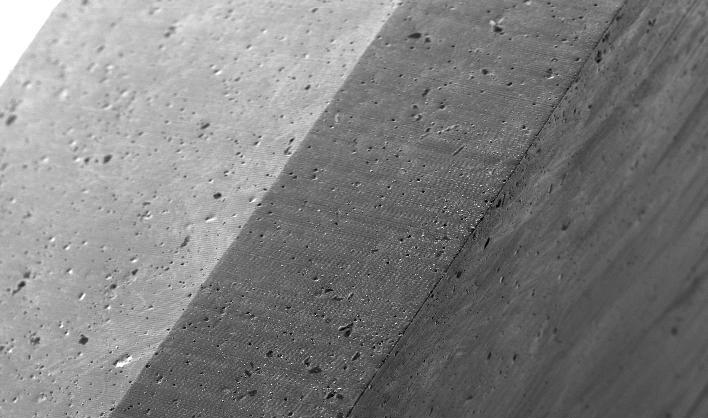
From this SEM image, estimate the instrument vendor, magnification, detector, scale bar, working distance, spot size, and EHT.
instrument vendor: FEI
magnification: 0.05 K X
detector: SE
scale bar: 500000 nm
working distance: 10.8 mm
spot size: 5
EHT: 20 kV